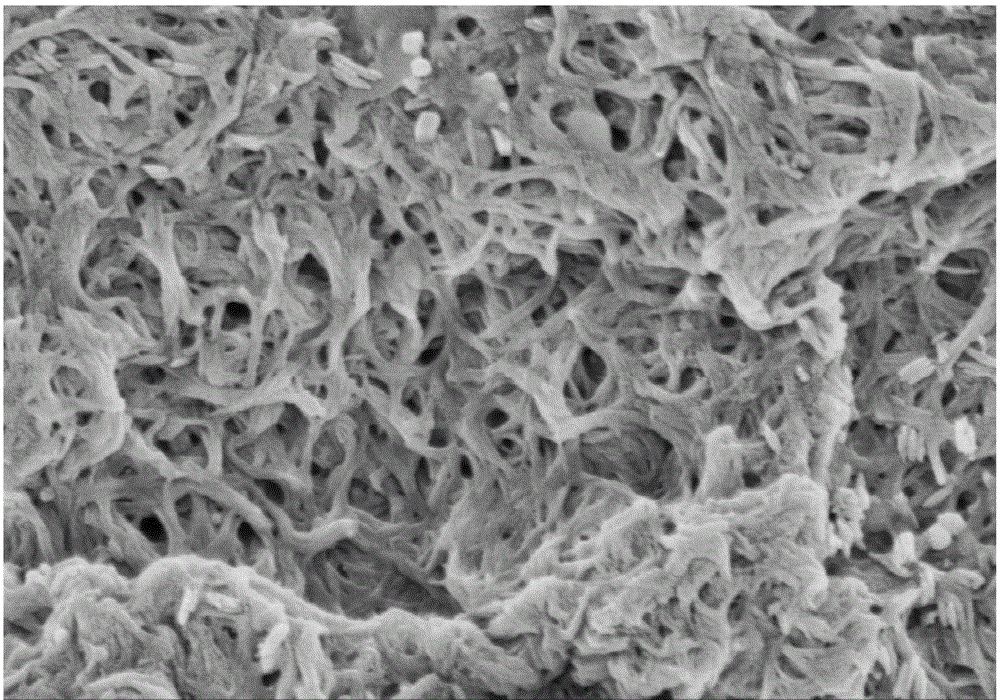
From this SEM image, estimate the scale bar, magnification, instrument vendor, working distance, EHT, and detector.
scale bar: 1000 nm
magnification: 50 K X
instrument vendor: Hitachi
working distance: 4.9 mm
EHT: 2 kV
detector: SE(M)